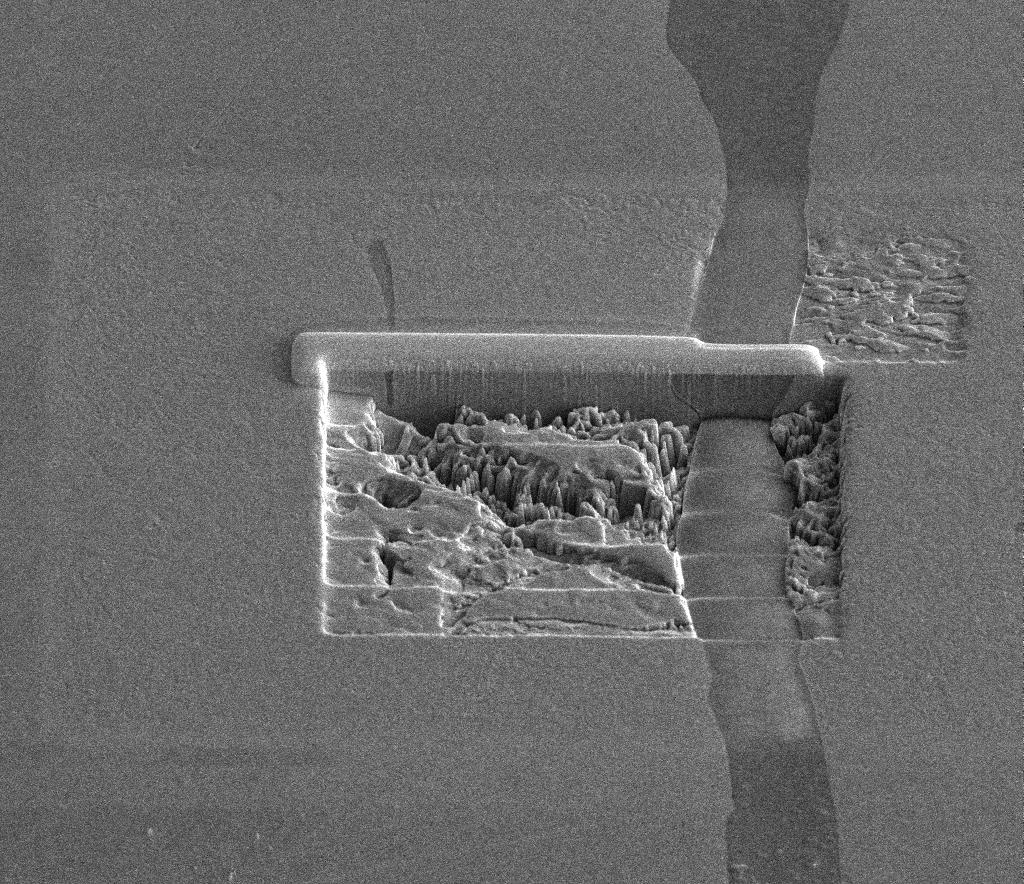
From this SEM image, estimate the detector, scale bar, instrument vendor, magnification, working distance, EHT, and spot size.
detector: ETD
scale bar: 20000 nm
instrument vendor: FEI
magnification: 2.5 K X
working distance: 10 mm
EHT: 10 kV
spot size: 4.5